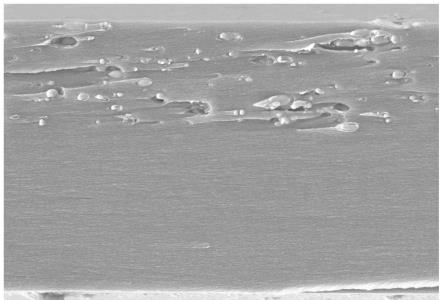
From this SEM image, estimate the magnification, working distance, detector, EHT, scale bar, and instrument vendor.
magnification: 3 K X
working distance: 13.4 mm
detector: SE(TUL)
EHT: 10 kV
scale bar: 10000 nm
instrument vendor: Hitachi